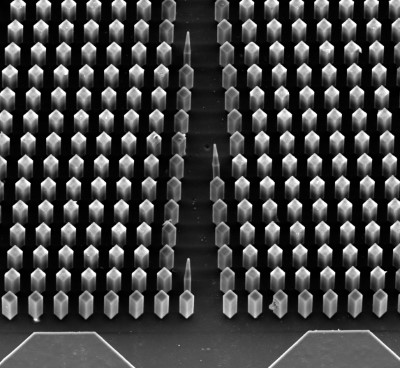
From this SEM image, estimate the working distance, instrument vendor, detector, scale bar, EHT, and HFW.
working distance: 32.07 mm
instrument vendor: Tescan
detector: SE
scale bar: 200000 nm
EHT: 20 kV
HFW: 713 µm